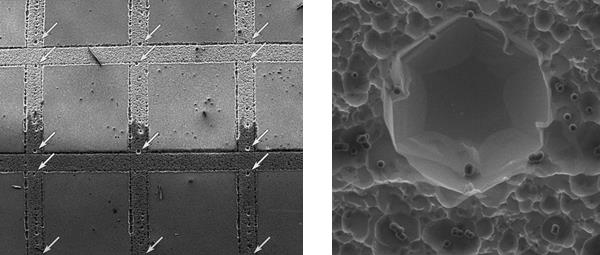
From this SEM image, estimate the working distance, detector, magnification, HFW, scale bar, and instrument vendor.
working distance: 11.32 mm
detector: SE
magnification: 0.09 K X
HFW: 4220 µm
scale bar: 1000000 nm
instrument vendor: Tescan